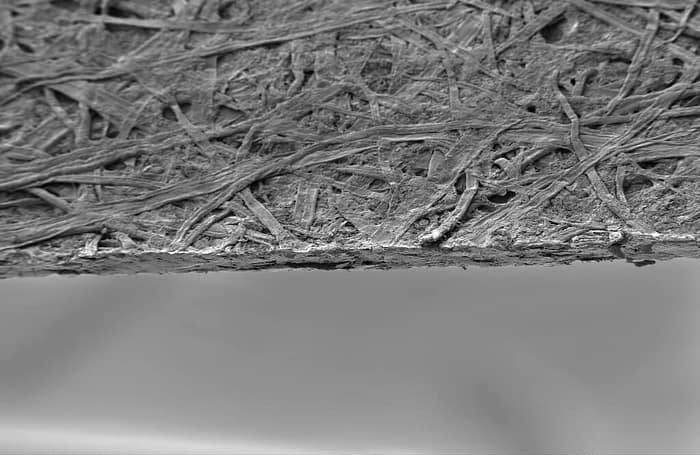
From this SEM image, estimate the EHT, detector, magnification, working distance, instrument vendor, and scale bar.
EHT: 3 kV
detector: SE2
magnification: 0.1 K X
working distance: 14.5 mm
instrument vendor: Zeiss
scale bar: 100000 nm